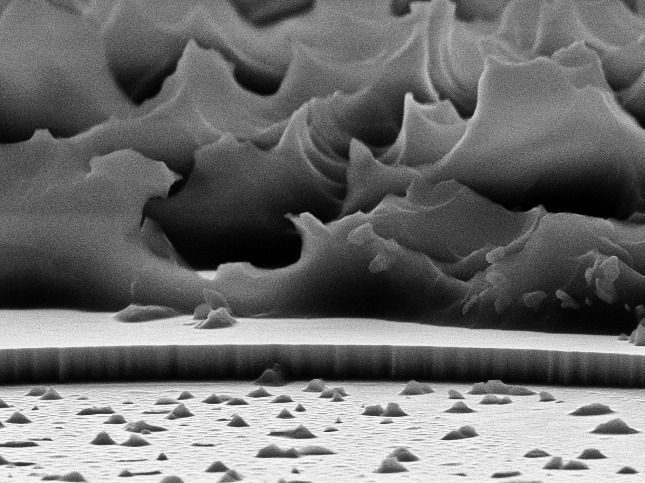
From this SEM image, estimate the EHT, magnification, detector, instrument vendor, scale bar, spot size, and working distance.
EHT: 10 kV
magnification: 16.126 K X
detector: SE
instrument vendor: FEI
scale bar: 1000 nm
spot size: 3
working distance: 11 mm